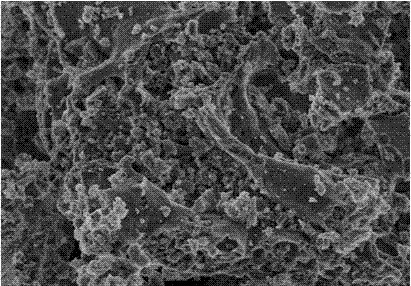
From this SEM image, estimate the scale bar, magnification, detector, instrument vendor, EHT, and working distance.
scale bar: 5000 nm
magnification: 10 K X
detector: SE(M)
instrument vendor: Hitachi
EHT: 3 kV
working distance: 7.3 mm